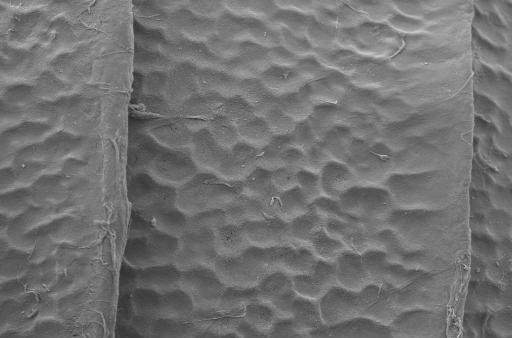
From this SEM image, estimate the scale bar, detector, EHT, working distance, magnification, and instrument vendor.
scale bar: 10000 nm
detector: SE2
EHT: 5 kV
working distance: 5.6 mm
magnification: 4.49 K X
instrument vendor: Zeiss